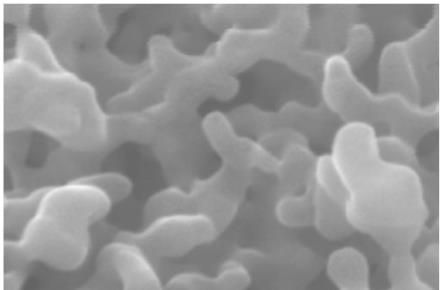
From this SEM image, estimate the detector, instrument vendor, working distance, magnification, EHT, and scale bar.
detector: InLens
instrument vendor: Zeiss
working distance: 6.2 mm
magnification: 100 K X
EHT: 20 kV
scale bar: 10 nm